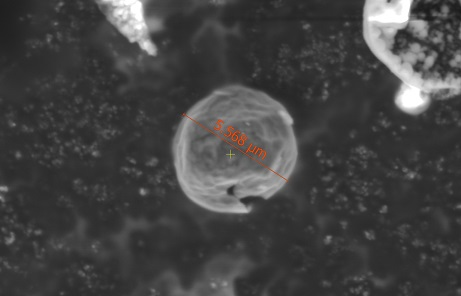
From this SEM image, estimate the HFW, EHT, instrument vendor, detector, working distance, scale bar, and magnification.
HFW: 20.7 µm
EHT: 5 kV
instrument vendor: FEI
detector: LVD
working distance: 10.6 mm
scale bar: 5000 nm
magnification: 10 K X